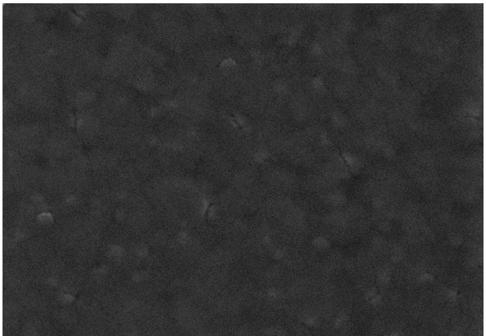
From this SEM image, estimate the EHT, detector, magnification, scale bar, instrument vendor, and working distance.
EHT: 5 kV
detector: SE(M)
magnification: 60 K X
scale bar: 500 nm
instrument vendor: Hitachi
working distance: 4.8 mm